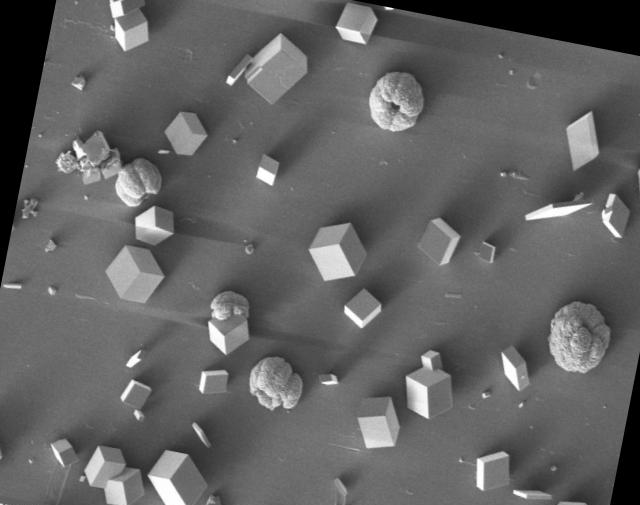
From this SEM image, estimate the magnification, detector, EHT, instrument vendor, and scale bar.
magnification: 0.4 K X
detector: SE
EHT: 5 kV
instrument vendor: Tescan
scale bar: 200000 nm